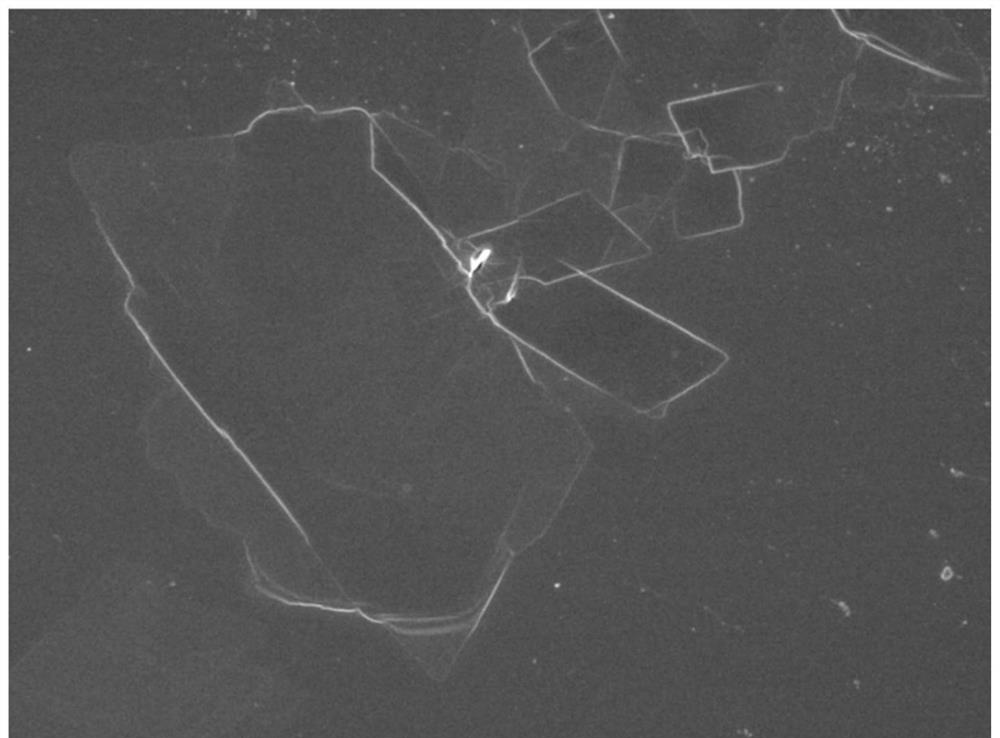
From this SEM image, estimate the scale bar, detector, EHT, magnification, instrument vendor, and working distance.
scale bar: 10000 nm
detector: SEI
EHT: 5 kV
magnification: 2.7 K X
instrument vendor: JEOL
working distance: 8.4 mm